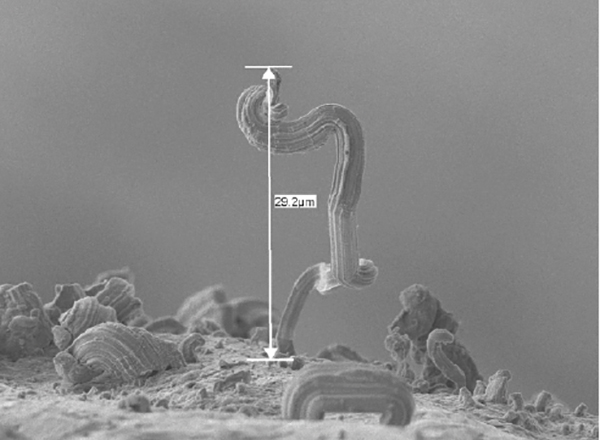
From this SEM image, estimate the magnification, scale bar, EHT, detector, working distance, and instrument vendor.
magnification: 2 K X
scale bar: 10000 nm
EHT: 5 kV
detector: LEI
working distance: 18 mm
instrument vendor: JEOL